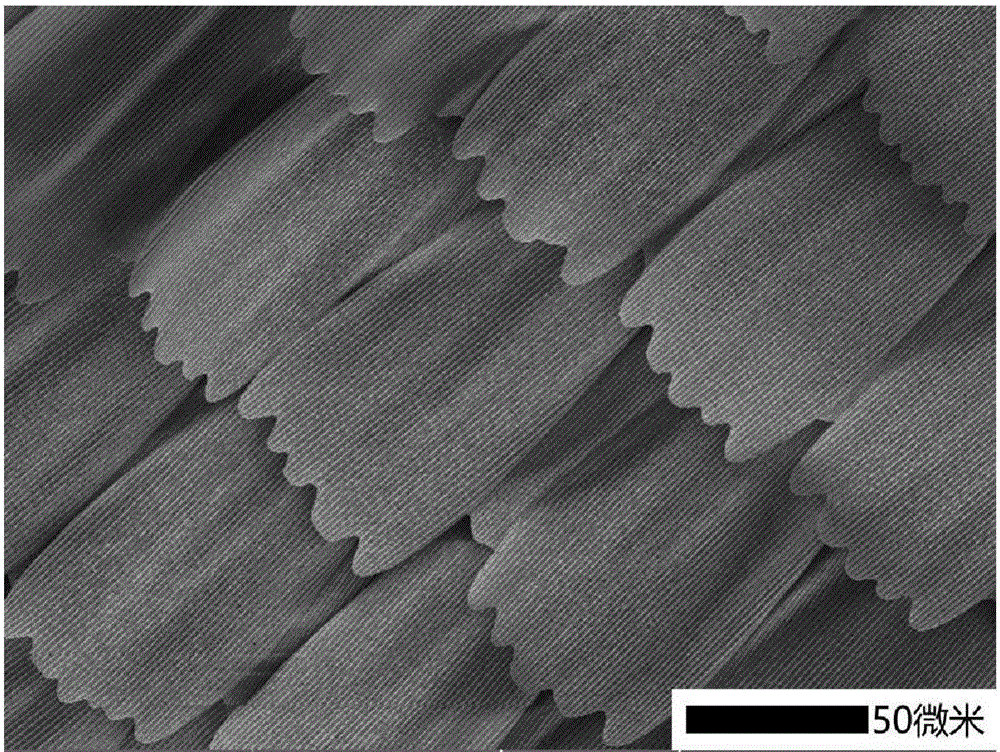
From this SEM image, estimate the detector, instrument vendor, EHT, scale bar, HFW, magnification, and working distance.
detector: SE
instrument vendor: Tescan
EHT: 5 kV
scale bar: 50000 nm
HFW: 277 µm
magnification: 0.748 K X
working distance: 6.06 mm